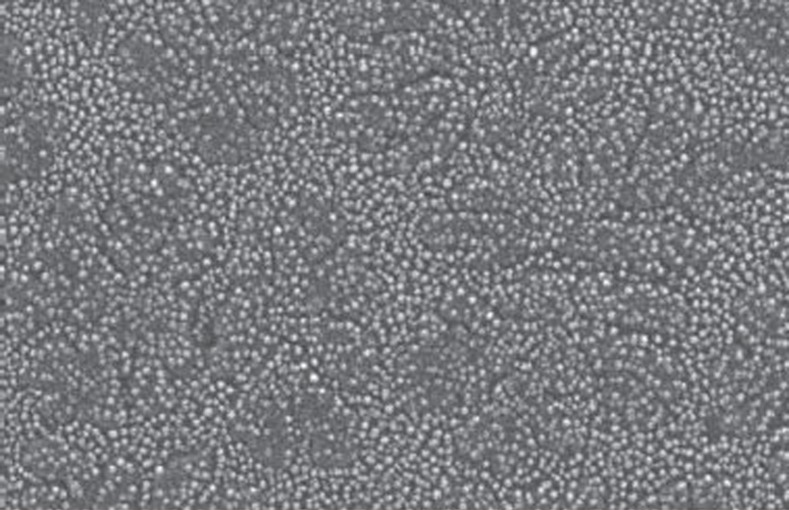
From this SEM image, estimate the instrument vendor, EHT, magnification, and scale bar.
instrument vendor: JEOL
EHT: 20 kV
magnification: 40 K X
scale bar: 500 nm